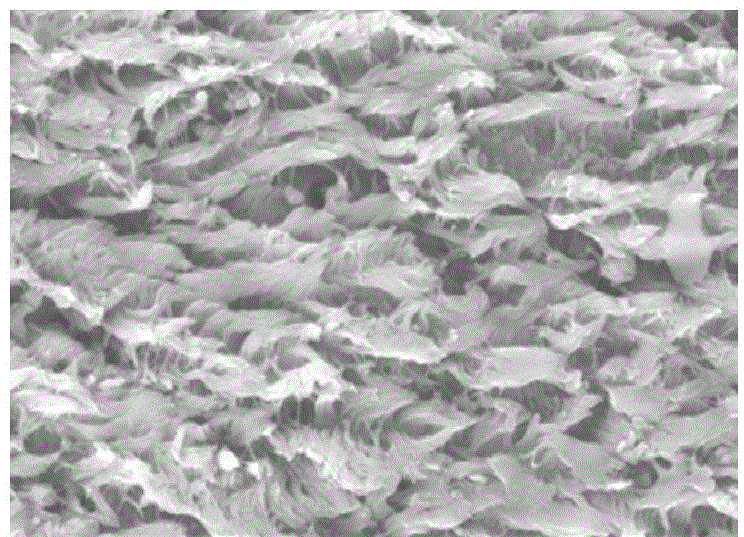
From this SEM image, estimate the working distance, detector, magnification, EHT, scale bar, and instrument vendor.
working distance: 24.3 mm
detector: SE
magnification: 2 K X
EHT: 20 kV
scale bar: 20000 nm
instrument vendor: Hitachi